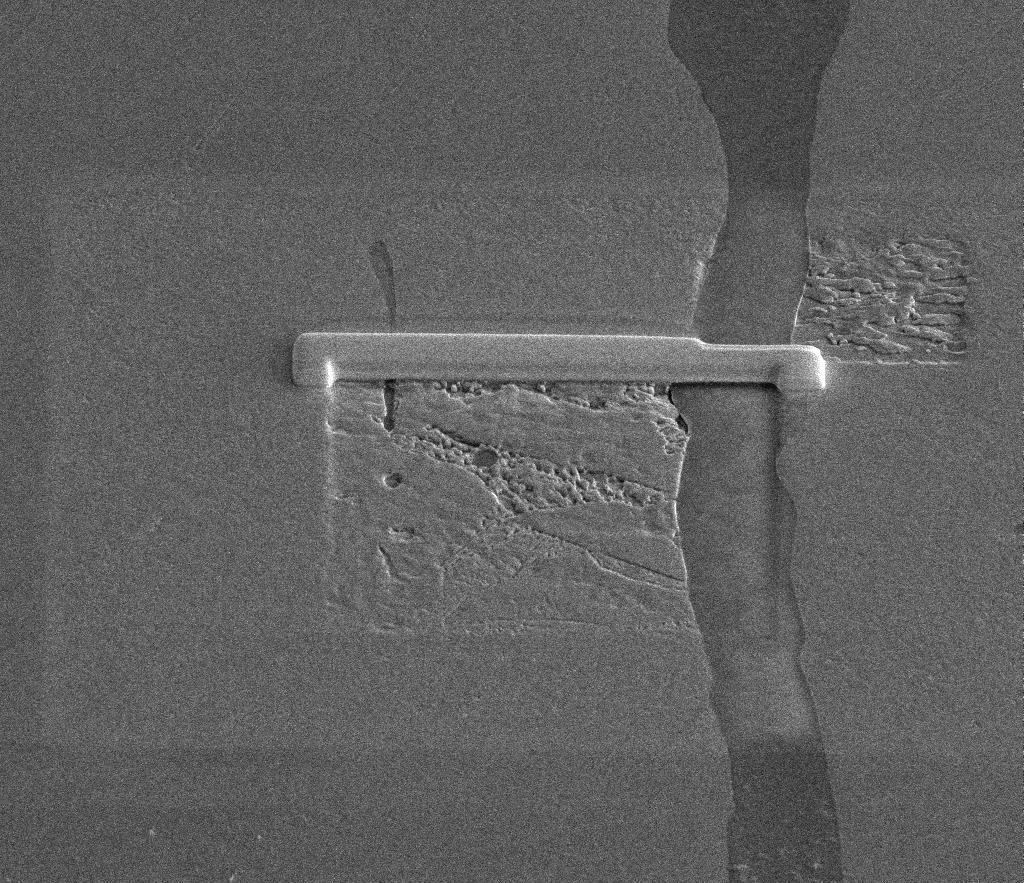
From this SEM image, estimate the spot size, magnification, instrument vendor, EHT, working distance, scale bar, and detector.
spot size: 4.5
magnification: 2.5 K X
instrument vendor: FEI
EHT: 10 kV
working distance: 10 mm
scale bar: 20000 nm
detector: ETD